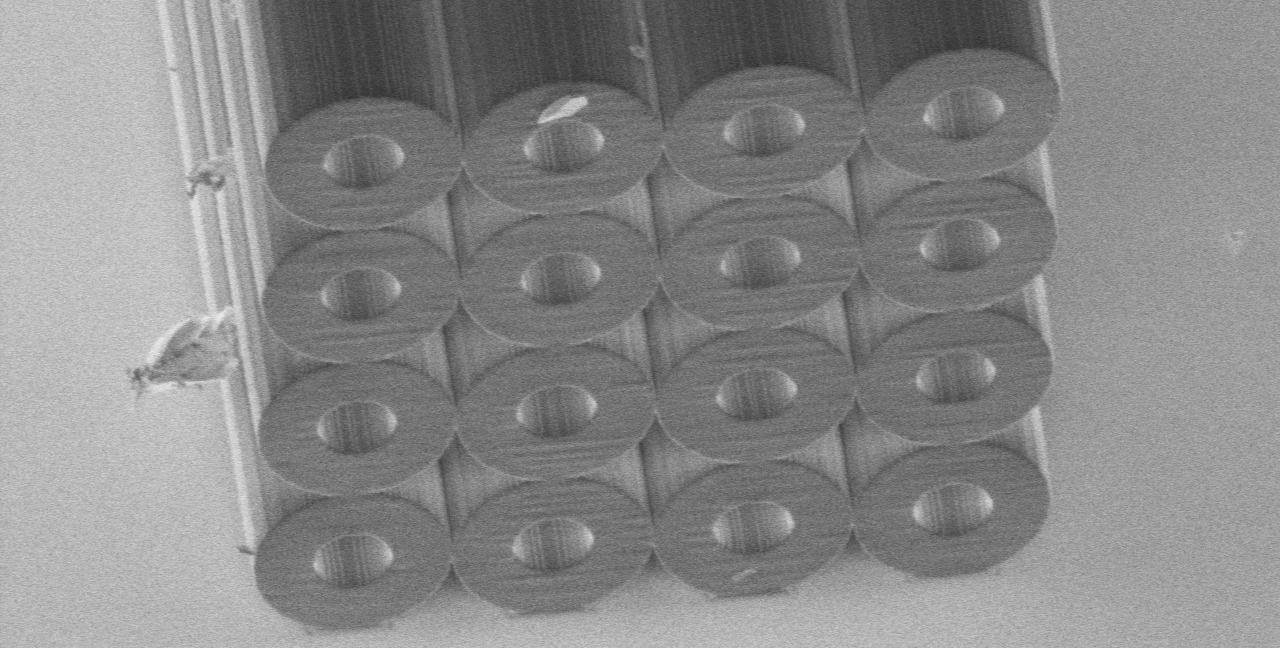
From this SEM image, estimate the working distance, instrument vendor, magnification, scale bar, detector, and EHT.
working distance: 0 mm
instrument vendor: Hitachi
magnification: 0.8 K X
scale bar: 50000 nm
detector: SE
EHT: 1 kV